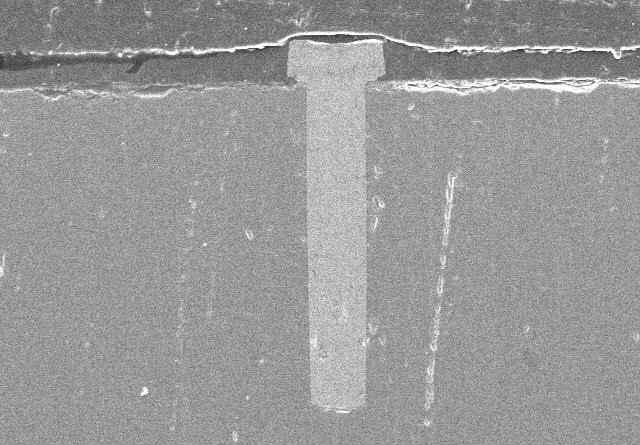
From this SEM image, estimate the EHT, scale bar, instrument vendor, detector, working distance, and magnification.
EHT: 10 kV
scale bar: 100000 nm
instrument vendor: Hitachi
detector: SE(M)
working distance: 5.8 mm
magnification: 0.45 K X